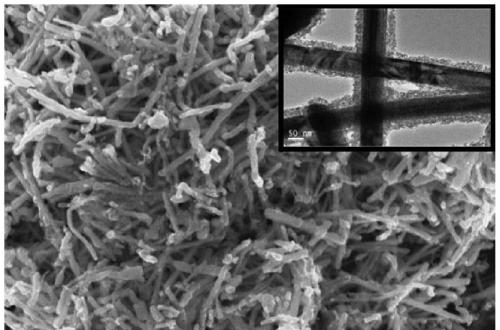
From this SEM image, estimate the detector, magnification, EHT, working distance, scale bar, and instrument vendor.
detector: InLens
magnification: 10 K X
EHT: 12 kV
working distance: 4.7 mm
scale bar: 1000 nm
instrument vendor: Zeiss